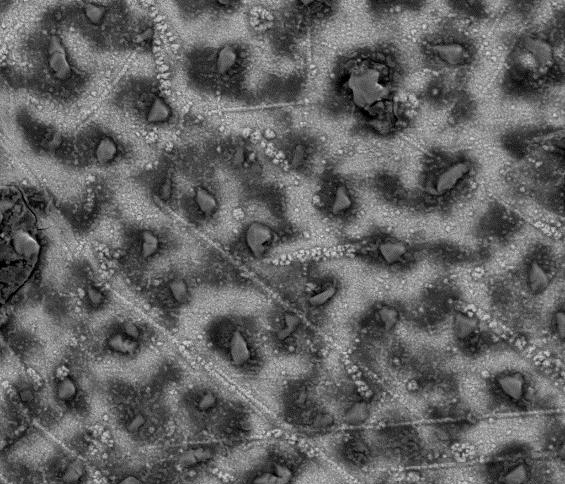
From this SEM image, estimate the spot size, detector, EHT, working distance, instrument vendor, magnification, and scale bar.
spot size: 4.5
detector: Mix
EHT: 15 kV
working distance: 10.6 mm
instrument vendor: FEI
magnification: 1 K X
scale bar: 50000 nm